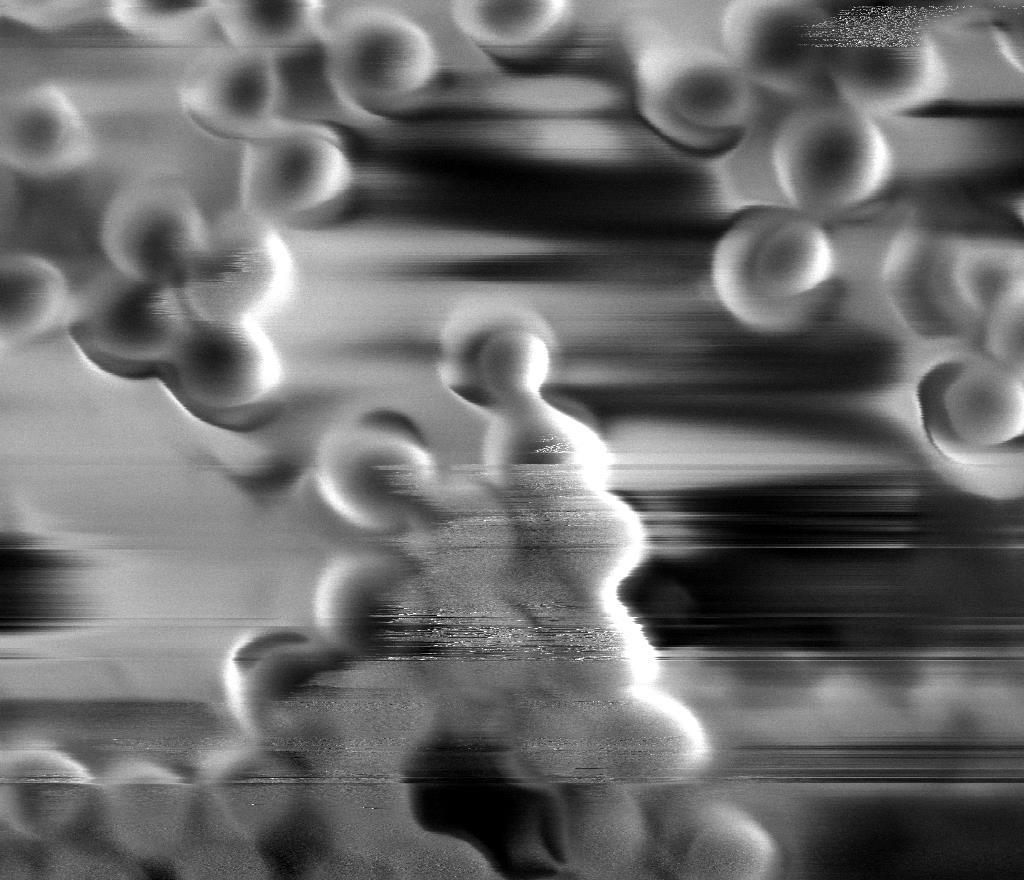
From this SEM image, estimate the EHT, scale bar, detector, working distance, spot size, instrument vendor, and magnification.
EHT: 4 kV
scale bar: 500 nm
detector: ETD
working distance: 5.6 mm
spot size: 4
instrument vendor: FEI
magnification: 100 K X